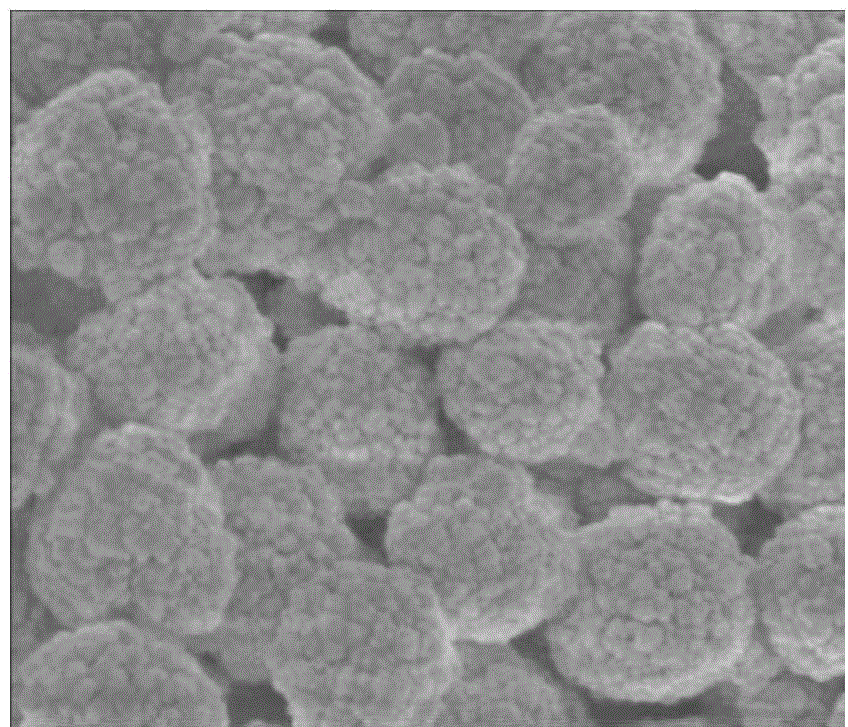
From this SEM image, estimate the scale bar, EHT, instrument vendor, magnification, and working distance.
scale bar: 500 nm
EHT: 3 kV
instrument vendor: FEI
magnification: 160 K X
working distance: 4.4 mm